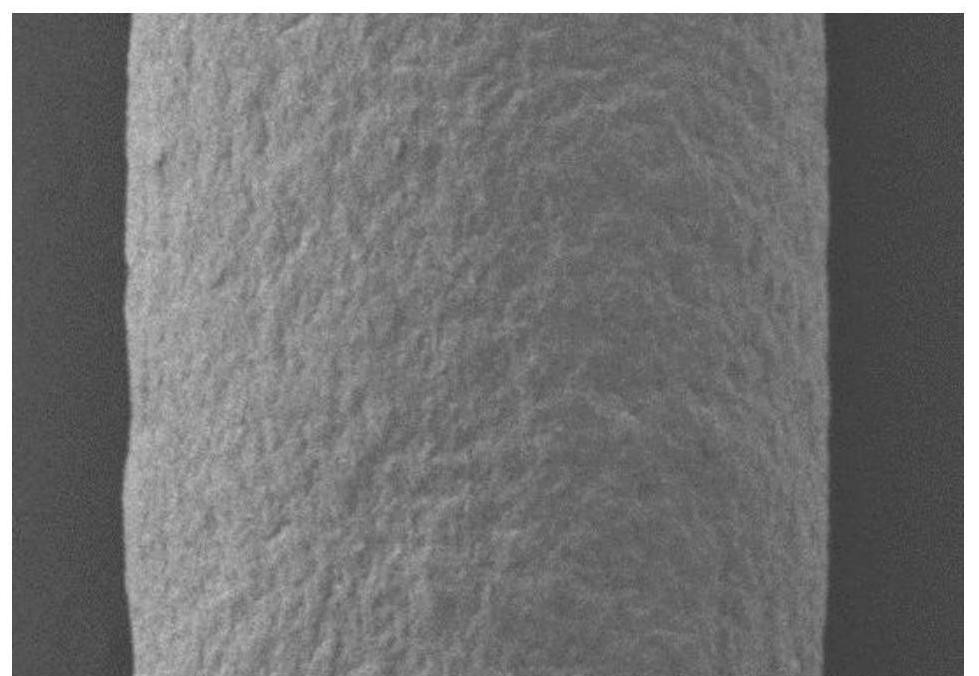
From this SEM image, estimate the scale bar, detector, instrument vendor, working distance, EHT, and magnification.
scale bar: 500000 nm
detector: LM(UL)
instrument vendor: Hitachi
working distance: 9.7 mm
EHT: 5 kV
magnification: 0.1 K X